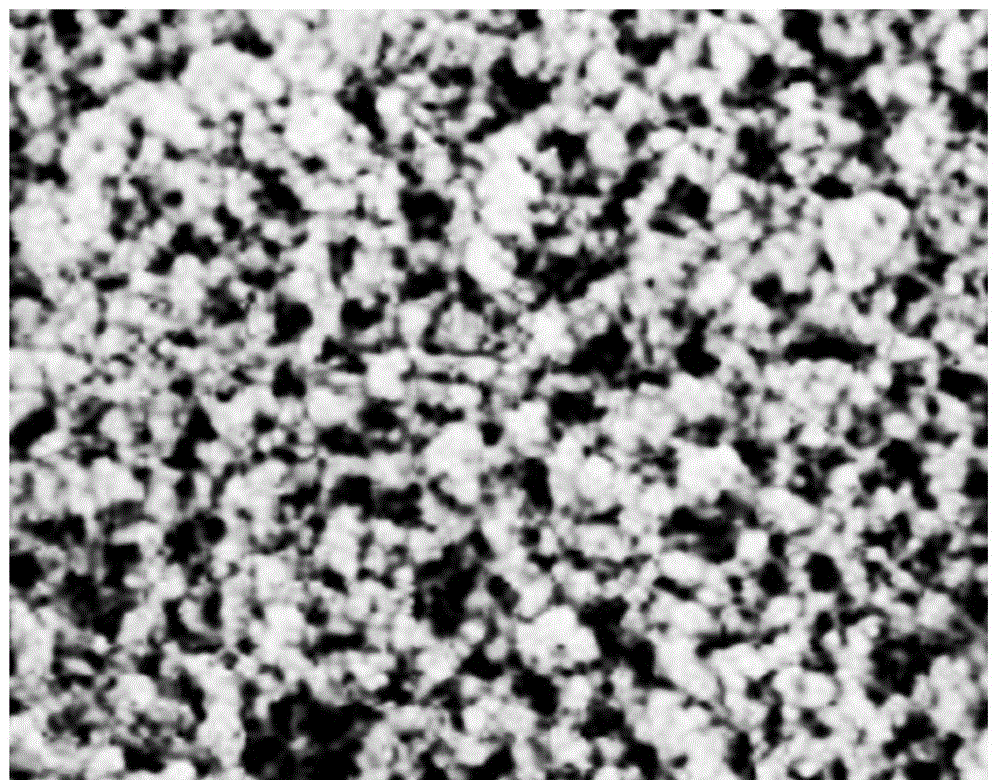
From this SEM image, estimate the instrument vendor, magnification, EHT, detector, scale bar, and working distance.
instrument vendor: JEOL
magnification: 40 K X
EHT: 15 kV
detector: LED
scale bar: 100 nm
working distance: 10.3 mm